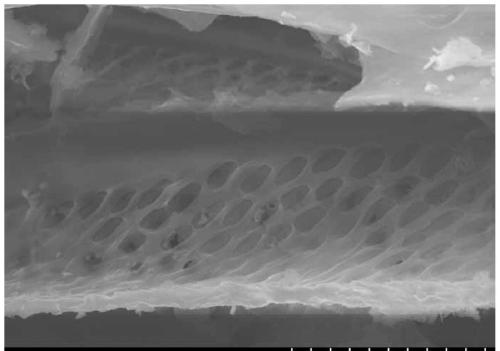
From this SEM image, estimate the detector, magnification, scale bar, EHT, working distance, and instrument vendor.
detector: SE(U)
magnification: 5 K X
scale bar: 10000 nm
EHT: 15 kV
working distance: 14.5 mm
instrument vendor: Hitachi